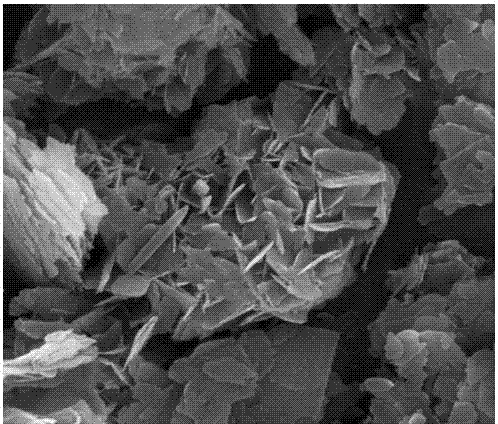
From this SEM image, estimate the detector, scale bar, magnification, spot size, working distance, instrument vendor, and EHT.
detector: ETD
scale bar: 3000 nm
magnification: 30 K X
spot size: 2.5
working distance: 10.9 mm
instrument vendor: FEI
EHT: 20 kV